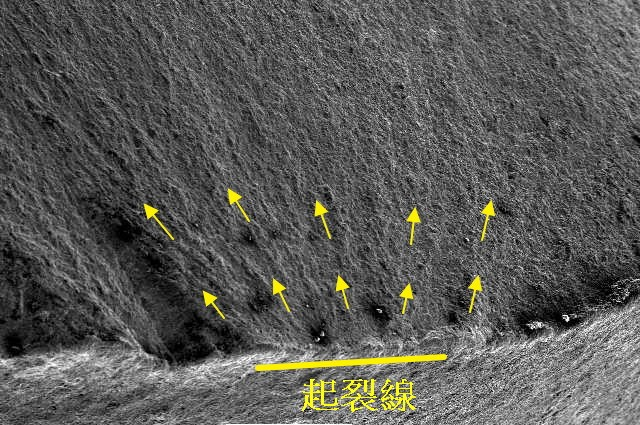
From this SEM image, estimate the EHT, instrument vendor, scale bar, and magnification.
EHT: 20 kV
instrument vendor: JEOL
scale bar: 1000000 nm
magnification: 0.025 K X